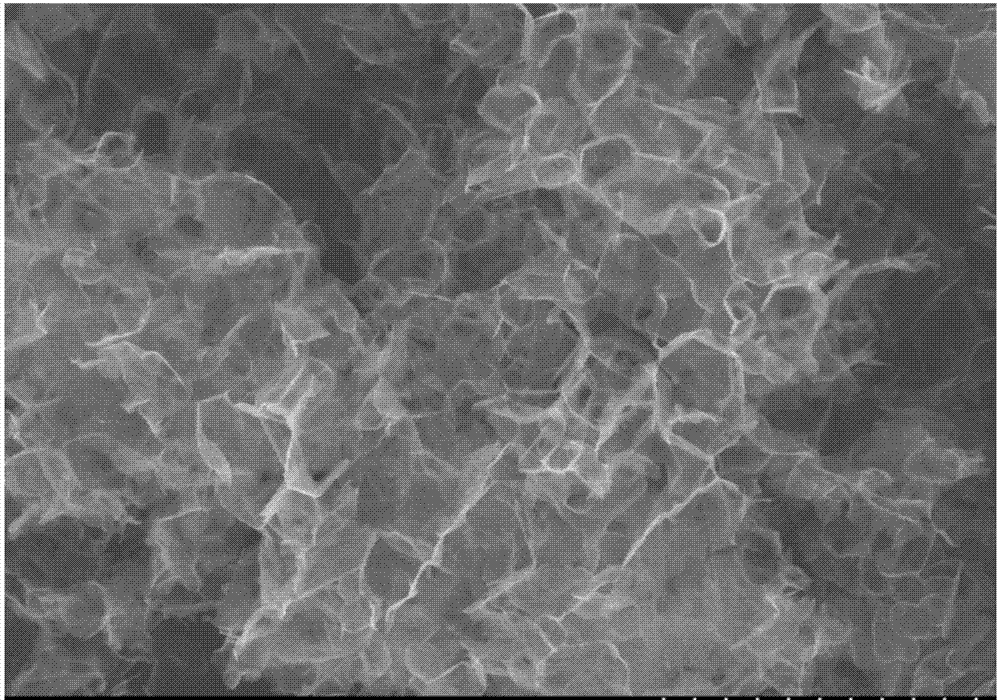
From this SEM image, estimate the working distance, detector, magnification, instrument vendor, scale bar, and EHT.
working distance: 10.5 mm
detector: SE(M)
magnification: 20 K X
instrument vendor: Hitachi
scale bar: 2000 nm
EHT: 5 kV